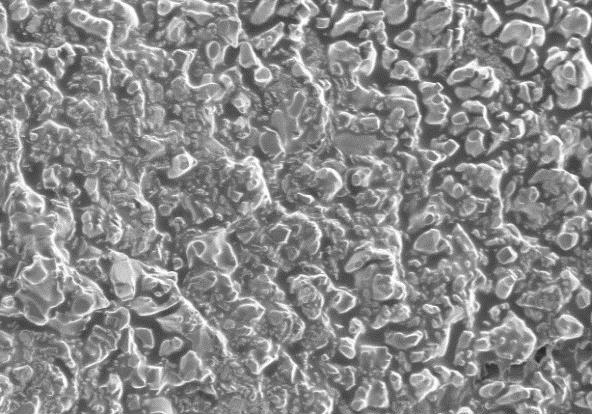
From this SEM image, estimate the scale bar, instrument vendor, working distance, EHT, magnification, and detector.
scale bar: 50000 nm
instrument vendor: Hitachi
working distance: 6.9 mm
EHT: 5 kV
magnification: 1 K X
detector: SE